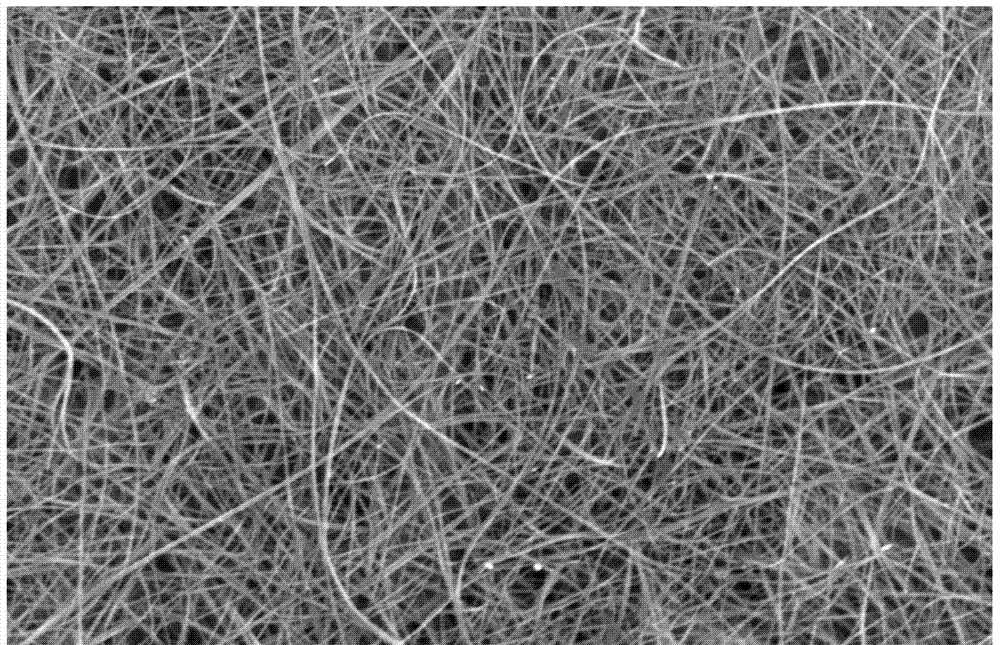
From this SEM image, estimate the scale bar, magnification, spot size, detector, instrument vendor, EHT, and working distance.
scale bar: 5000 nm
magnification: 5 K X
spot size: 4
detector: SE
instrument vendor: FEI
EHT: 25 kV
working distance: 4.9 mm